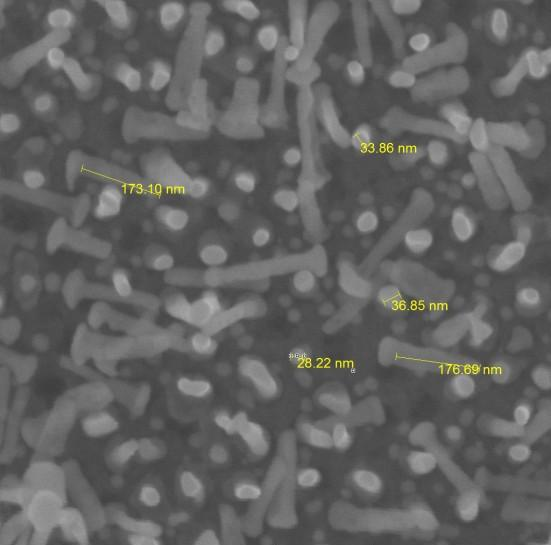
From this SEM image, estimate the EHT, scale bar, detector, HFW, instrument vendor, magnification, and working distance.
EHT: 15 kV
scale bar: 200 nm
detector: InBeam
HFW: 1.156 µm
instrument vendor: Tescan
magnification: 250 K X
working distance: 2.976 mm